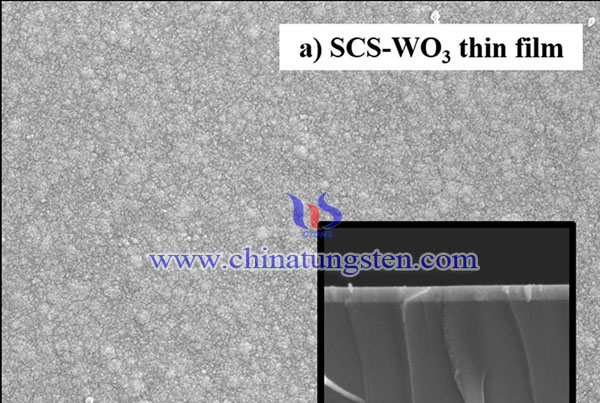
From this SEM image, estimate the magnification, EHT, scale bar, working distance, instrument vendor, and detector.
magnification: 30 K X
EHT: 15 kV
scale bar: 100 nm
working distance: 15 mm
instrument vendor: JEOL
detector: SEI